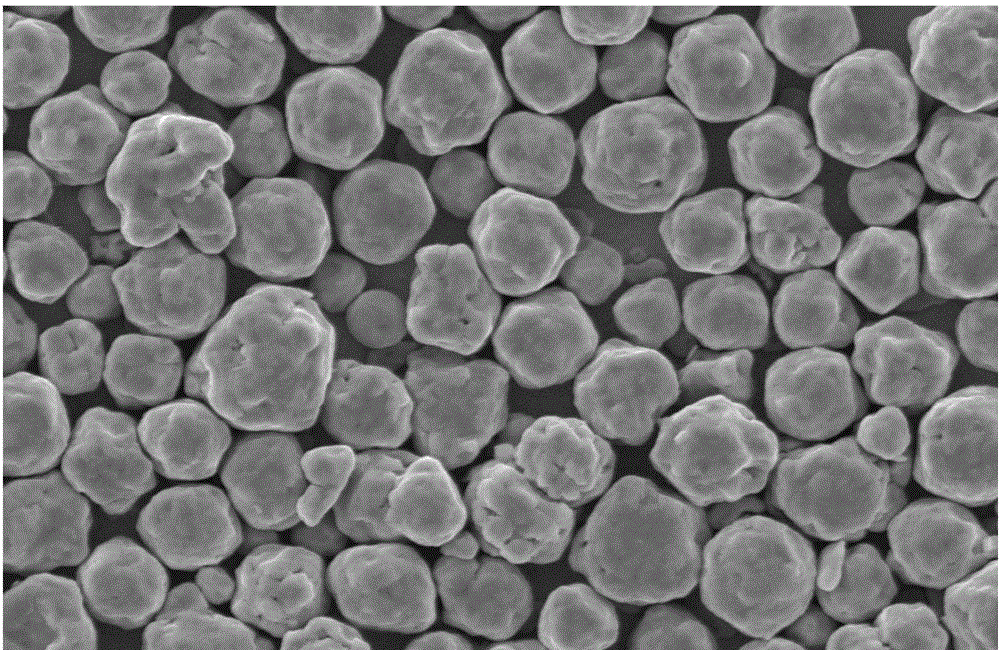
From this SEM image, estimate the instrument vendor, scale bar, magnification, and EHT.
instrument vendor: other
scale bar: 10000 nm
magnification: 2 K X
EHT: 20 kV